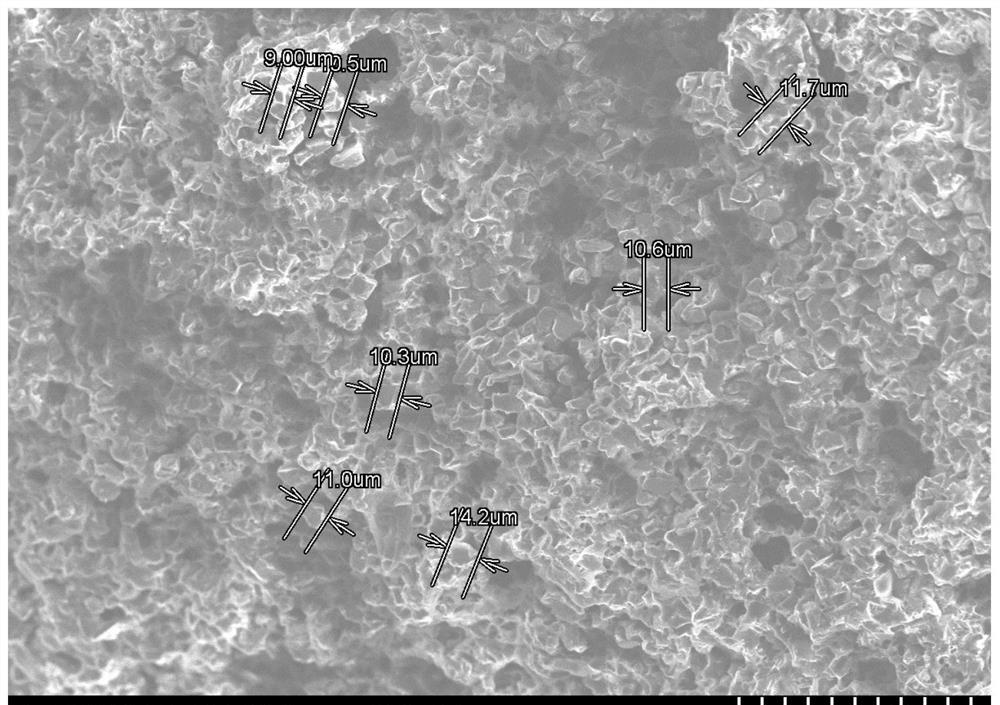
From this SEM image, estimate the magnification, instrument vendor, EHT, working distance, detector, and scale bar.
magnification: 0.3 K X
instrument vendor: Hitachi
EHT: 15 kV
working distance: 10.6 mm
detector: SE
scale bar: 100000 nm